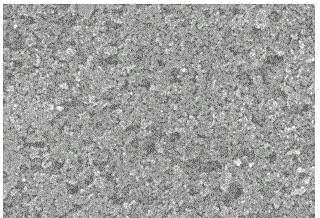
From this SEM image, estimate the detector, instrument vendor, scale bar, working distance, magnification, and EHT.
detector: SE(M)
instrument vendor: Hitachi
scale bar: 2000 nm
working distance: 8.9 mm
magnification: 20 K X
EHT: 15 kV